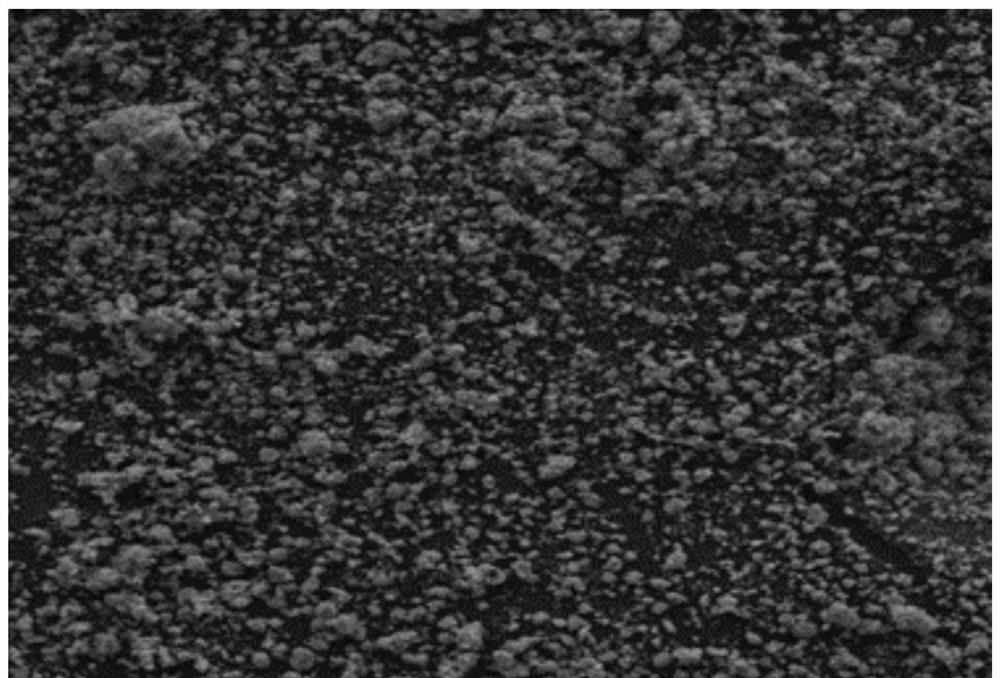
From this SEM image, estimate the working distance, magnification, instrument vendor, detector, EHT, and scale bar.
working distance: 15.99 mm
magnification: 0.5 K X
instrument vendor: Tescan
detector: SE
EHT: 20 kV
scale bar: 100000 nm